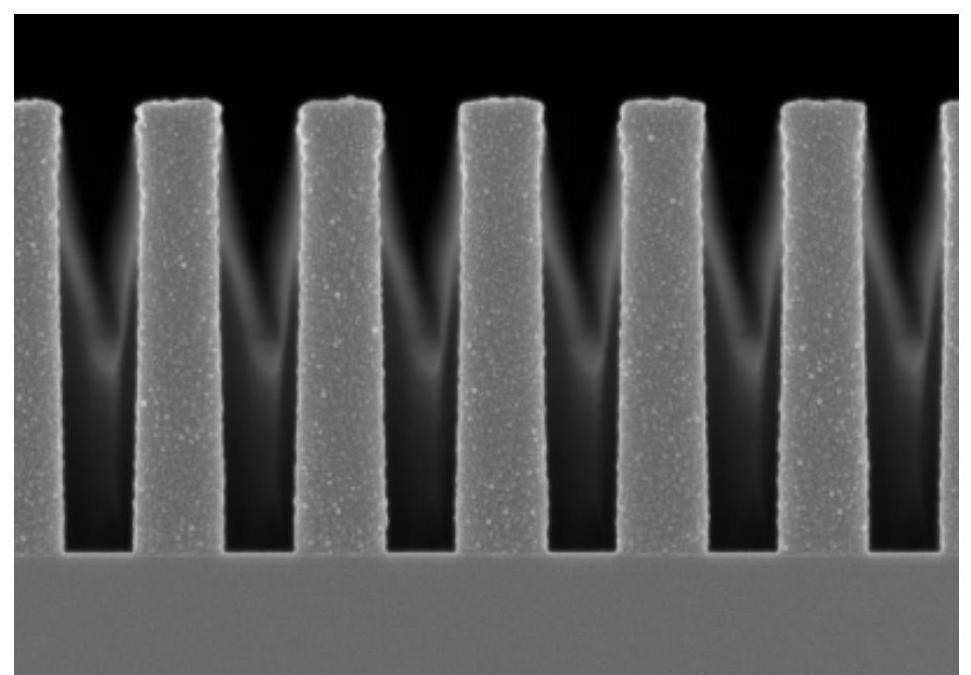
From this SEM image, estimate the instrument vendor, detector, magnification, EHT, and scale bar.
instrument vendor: Hitachi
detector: SE(U)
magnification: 35 K X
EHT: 2 kV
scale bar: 1000 nm